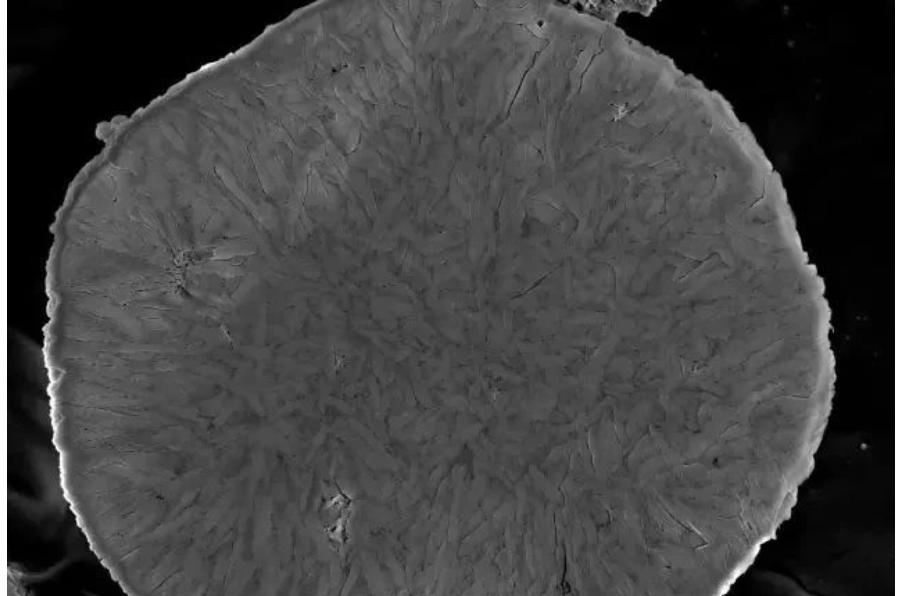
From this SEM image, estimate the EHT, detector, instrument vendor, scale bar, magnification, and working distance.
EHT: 5 kV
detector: InLens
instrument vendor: Zeiss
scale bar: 1000 nm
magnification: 10 K X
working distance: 3.4 mm